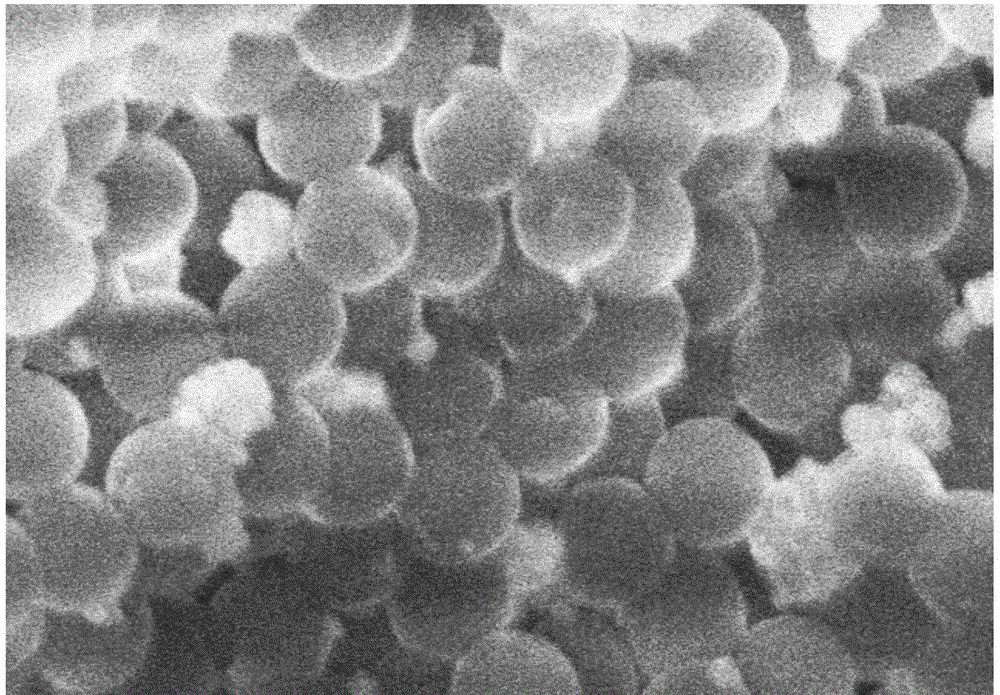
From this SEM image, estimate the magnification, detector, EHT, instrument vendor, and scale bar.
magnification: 100 K X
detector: SE(M,LA0)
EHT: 5 kV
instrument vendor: Hitachi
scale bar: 500 nm